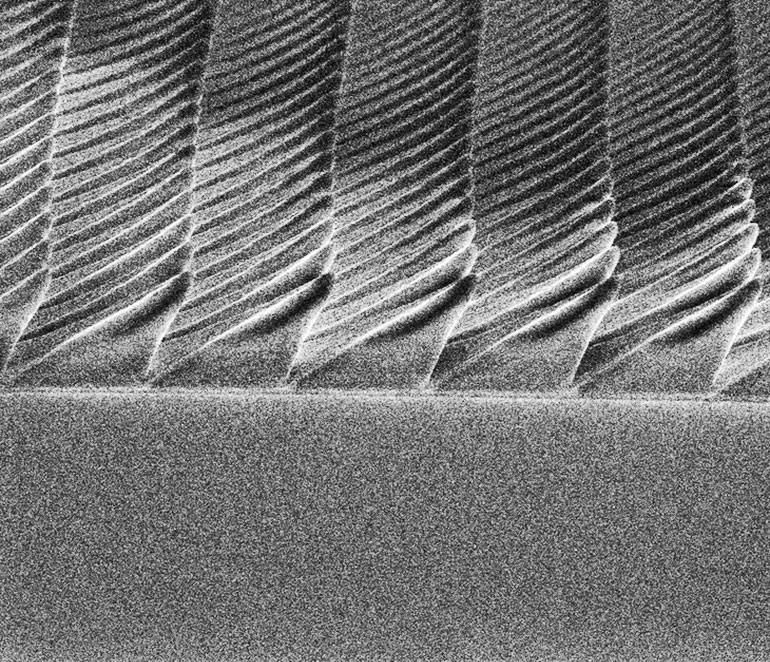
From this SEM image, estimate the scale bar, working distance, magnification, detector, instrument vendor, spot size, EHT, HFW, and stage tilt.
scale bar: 5000 nm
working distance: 4.8 mm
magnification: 11 K X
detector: ETD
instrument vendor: FEI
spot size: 2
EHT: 5 kV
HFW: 27.1 µm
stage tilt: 45°